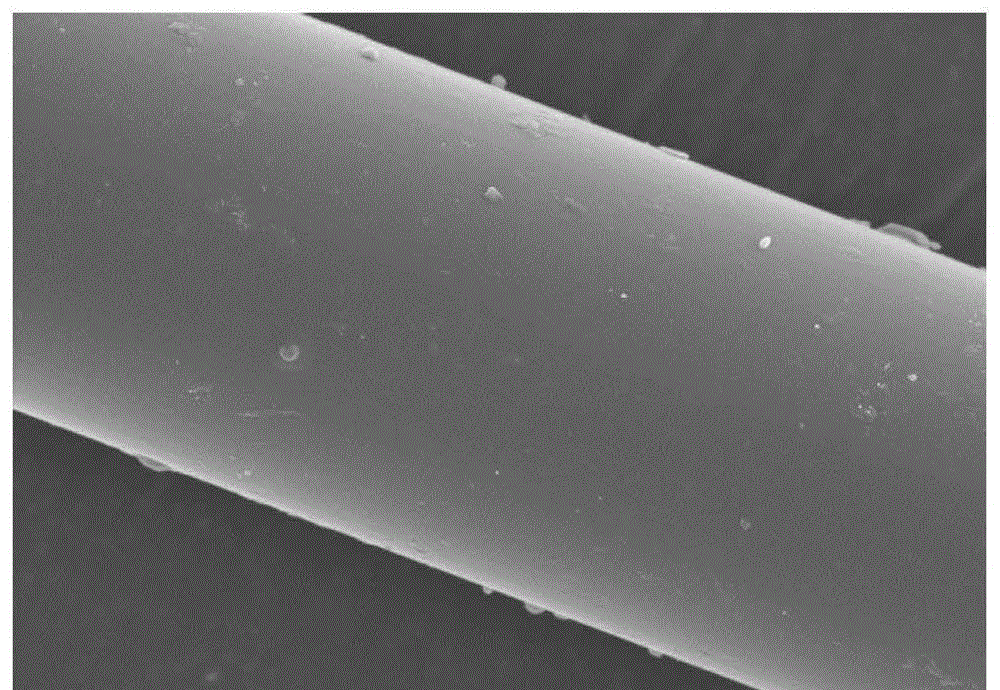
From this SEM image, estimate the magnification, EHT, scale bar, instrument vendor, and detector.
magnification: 5 K X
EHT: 5 kV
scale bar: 10000 nm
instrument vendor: Hitachi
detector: SE(M)